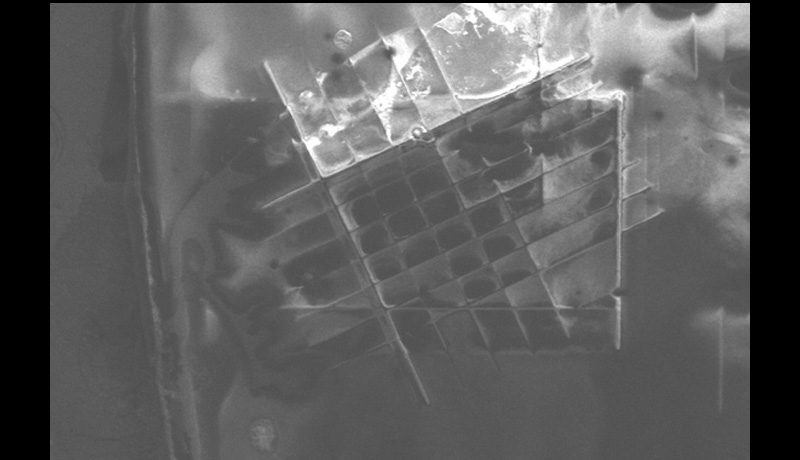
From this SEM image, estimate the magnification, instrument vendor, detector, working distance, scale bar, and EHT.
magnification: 0.075 K X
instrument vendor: JEOL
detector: LED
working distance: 10 mm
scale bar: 100000 nm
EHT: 5 kV